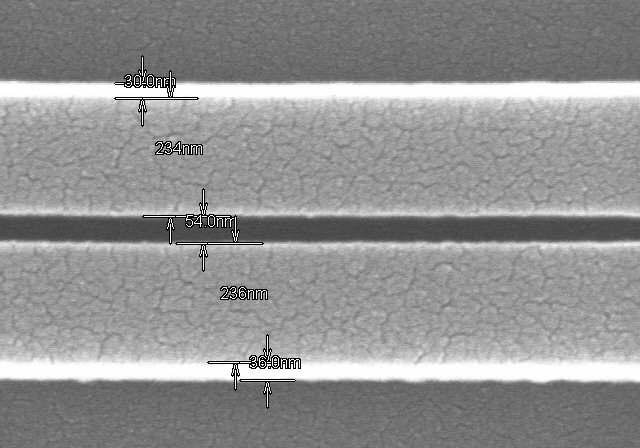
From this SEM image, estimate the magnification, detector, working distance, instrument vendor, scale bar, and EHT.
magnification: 100 K X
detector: SE(M)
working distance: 11.2 mm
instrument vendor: Hitachi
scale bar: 500 nm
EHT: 15 kV